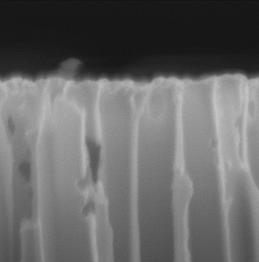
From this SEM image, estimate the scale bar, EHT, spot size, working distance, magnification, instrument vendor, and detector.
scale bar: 500 nm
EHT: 5 kV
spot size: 3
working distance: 5.2 mm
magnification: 100 K X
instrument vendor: FEI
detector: TLD